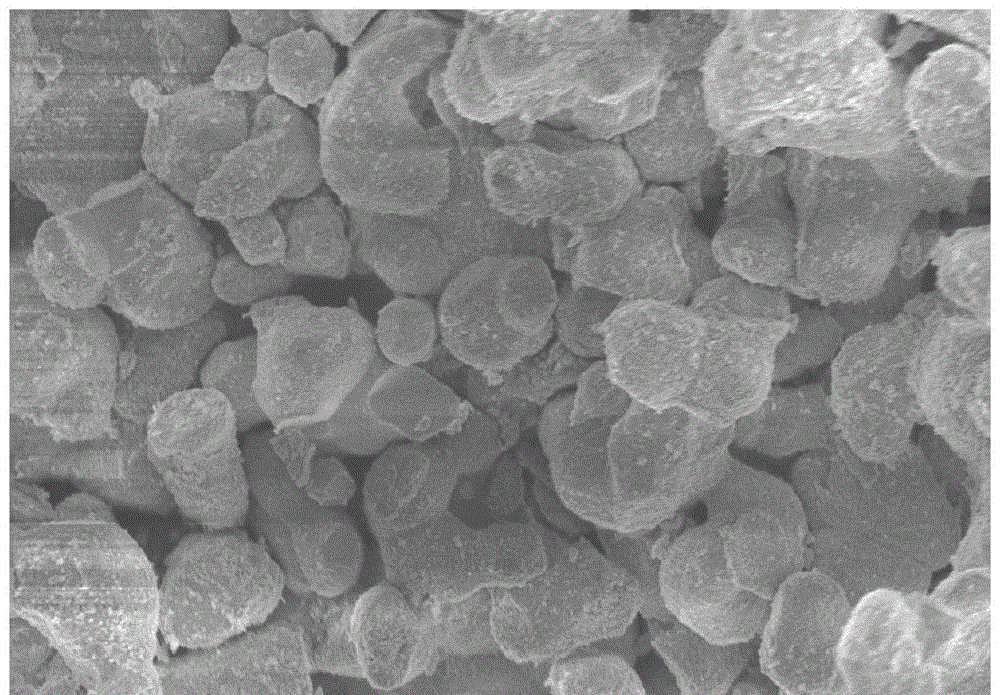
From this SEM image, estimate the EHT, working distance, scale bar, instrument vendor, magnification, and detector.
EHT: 5 kV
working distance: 8.3 mm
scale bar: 10000 nm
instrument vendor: Hitachi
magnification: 3 K X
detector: SE(M)